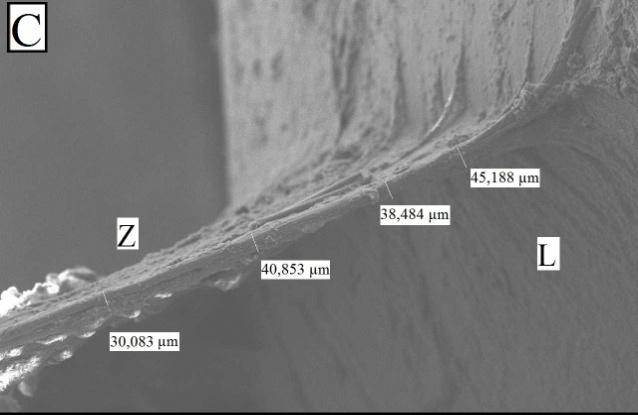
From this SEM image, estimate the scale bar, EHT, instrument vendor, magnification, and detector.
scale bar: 100000 nm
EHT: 15 kV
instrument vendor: JEOL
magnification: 0.1 K X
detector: SEI